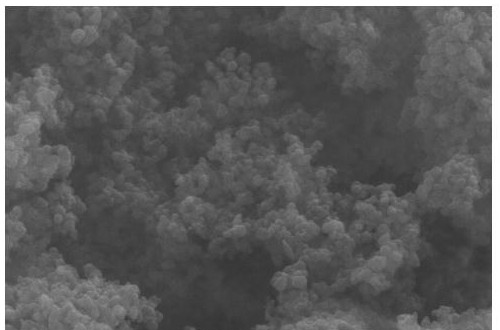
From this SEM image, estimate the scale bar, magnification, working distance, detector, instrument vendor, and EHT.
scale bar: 1000 nm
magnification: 32.43 K X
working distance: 13 mm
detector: SE2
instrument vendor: Zeiss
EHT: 10 kV